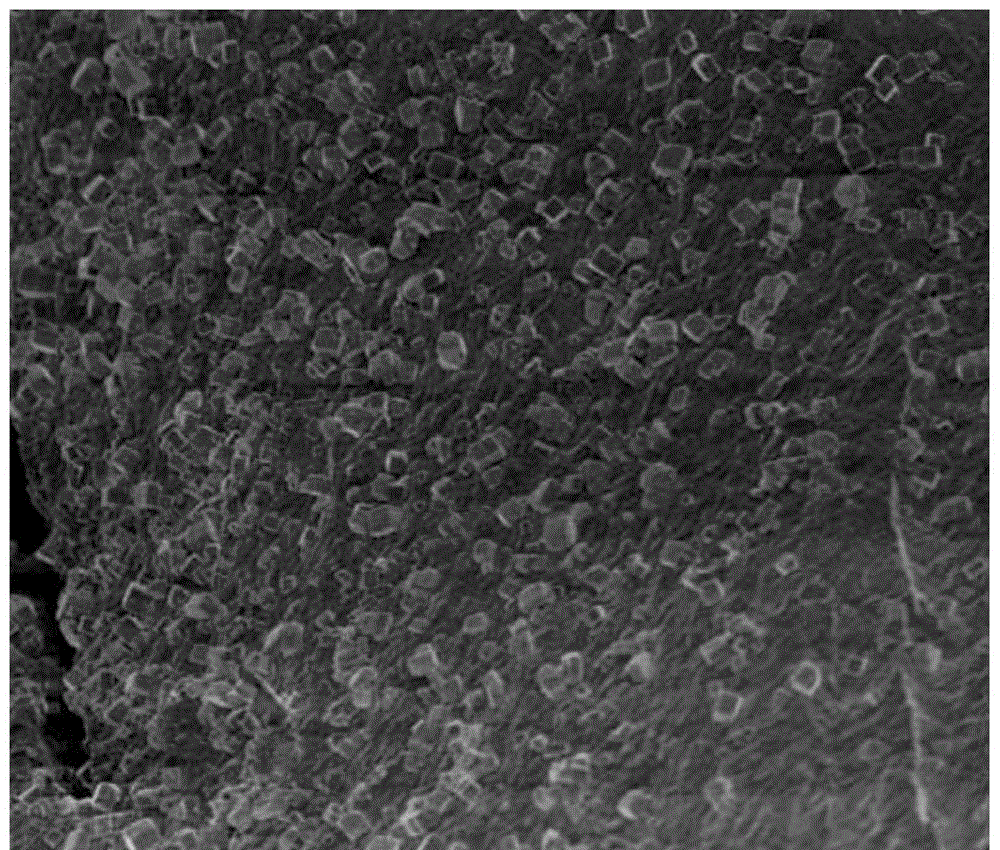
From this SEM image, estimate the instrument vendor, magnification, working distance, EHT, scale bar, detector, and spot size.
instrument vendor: FEI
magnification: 10 K X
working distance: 4.8 mm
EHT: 1 kV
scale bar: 5000 nm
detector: TLD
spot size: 3